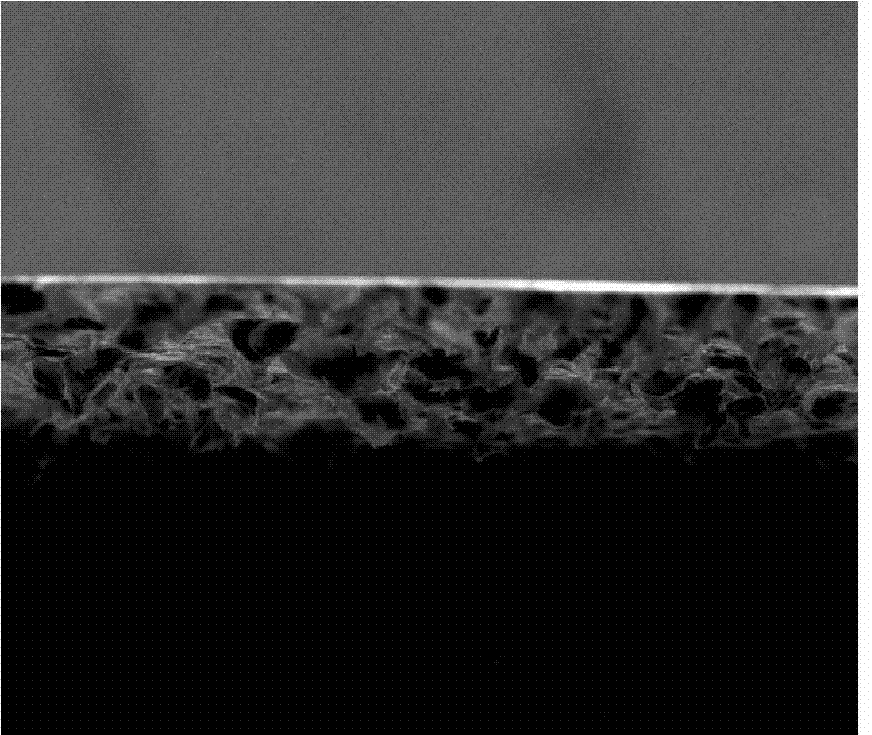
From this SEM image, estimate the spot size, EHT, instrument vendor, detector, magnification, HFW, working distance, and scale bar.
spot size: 3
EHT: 20 kV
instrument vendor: FEI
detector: ETD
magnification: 0.4 K X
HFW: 746 µm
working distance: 11.7 mm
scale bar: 300000 nm